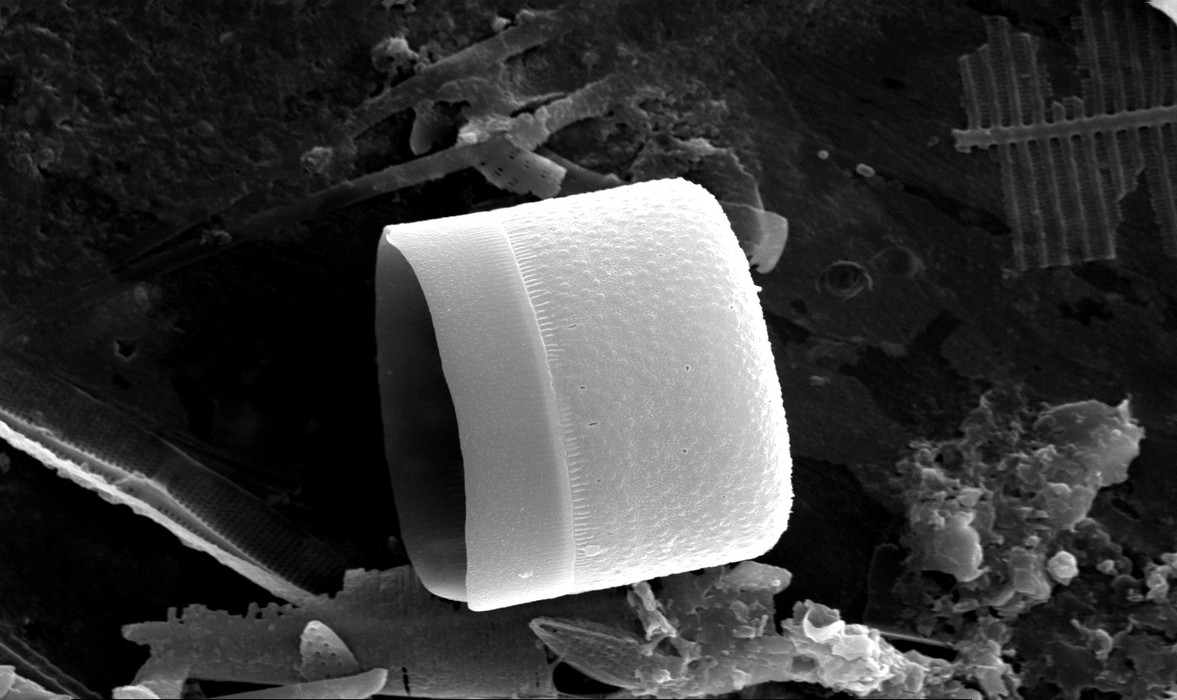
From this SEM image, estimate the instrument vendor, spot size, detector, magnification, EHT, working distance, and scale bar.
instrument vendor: FEI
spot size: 4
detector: SE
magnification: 5 K X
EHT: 25 kV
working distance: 9.9 mm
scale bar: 10000 nm